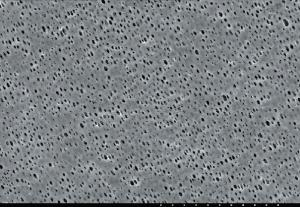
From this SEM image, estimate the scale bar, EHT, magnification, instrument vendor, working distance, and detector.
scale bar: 30000 nm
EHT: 15 kV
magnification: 1 K X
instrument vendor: JEOL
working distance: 32.7 mm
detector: SE